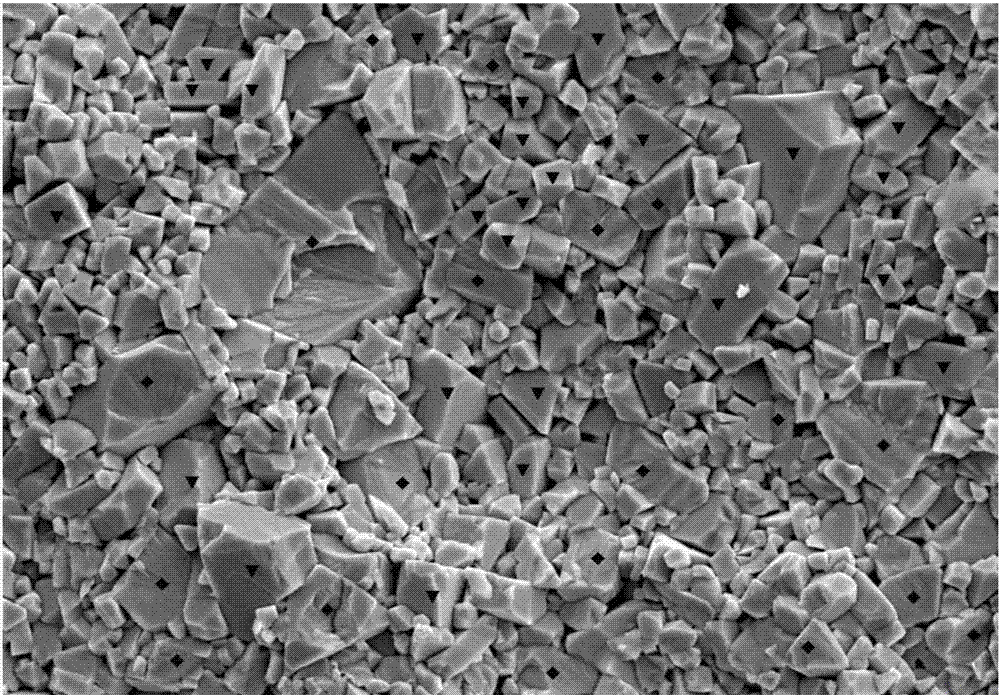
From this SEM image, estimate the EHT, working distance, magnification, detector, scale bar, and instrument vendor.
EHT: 15 kV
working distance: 20.3 mm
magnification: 3 K X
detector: SE2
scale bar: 1000 nm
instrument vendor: Zeiss